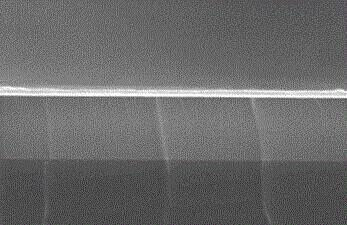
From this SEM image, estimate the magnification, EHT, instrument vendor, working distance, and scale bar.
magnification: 45 K X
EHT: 5 kV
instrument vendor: Hitachi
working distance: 8.7 mm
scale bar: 1000 nm